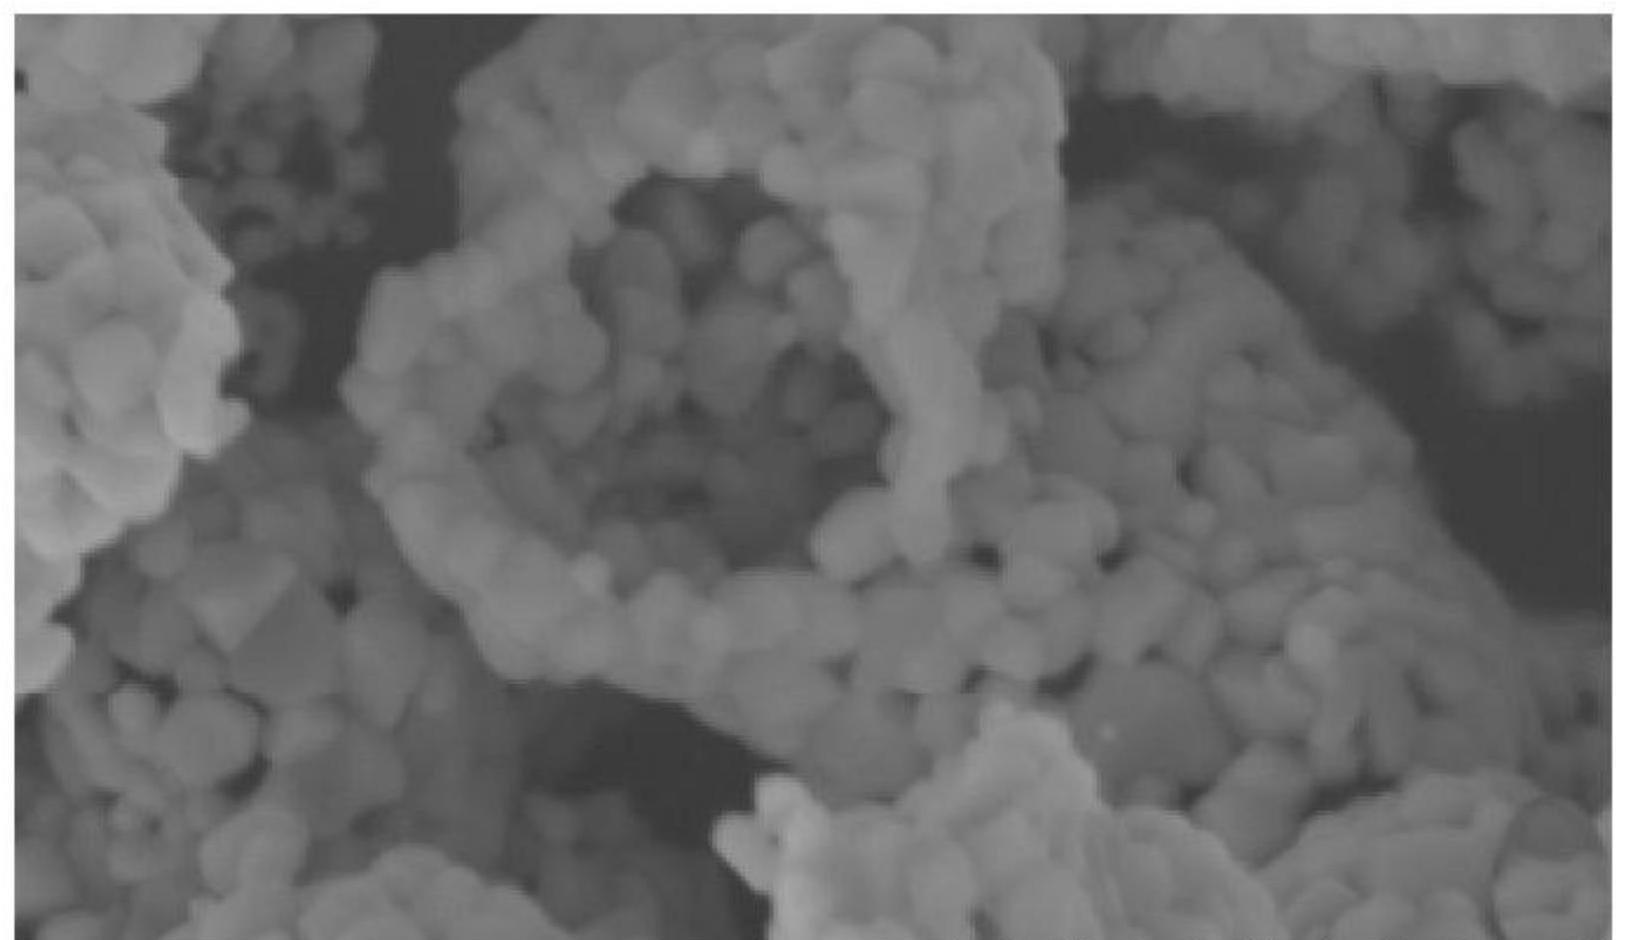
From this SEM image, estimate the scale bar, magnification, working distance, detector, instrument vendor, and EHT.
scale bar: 1000 nm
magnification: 50 K X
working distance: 5.4 mm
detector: SE(L)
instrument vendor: Hitachi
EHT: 5 kV